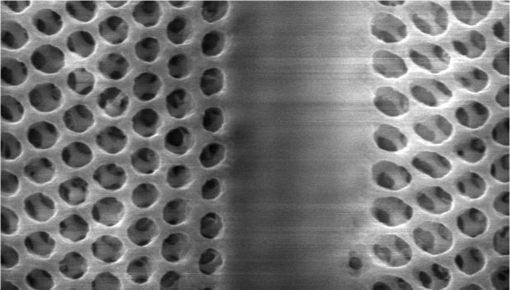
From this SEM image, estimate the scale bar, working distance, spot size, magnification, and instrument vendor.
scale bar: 2000 nm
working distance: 9 mm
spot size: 1.4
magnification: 12.8 K X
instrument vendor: FEI